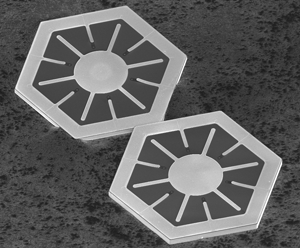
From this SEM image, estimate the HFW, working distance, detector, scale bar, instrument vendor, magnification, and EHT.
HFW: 829 µm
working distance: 5.3 mm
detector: TLD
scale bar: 300000 nm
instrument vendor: FEI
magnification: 0.18 K X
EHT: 5 kV